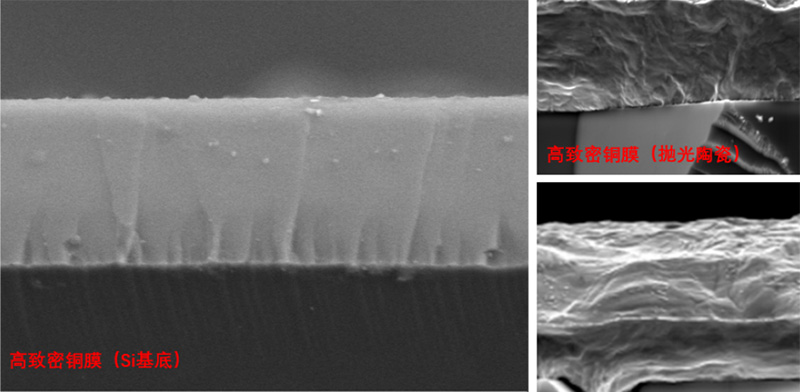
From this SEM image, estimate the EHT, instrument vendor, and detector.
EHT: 15 kV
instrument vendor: Zeiss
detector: SE2